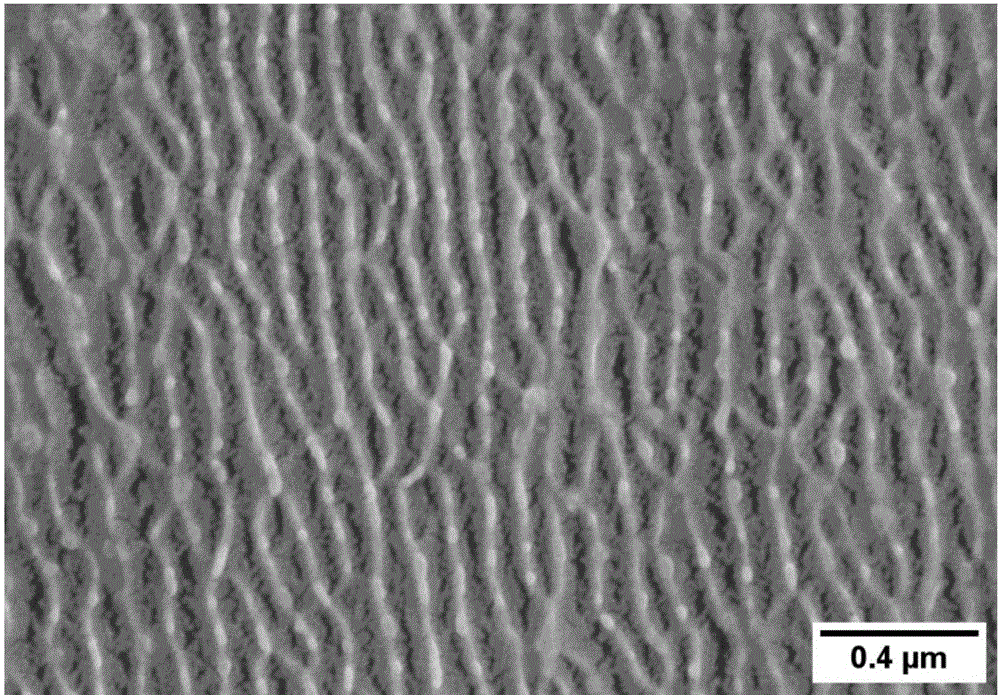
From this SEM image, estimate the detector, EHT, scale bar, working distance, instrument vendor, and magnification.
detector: SE(M)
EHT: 3 kV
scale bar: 1000 nm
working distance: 10.5 mm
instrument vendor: Hitachi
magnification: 50 K X